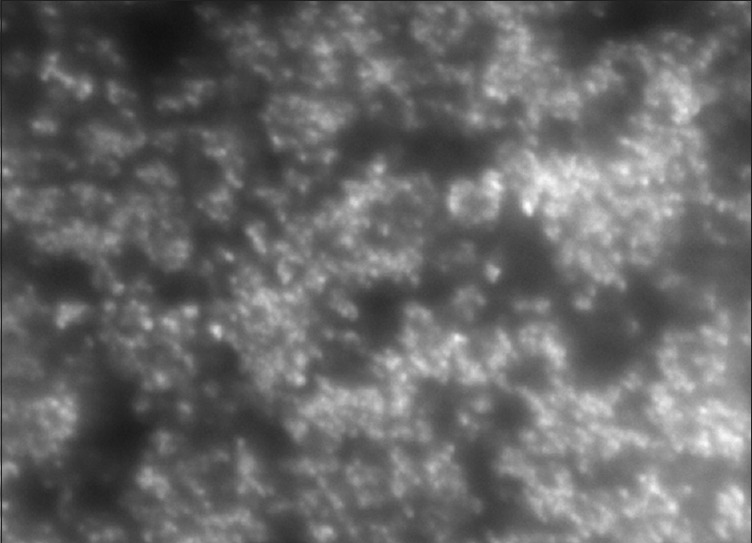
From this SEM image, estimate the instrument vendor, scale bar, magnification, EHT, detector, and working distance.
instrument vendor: Hitachi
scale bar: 10000 nm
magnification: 4 K X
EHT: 25 kV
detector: SE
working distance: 10.8 mm